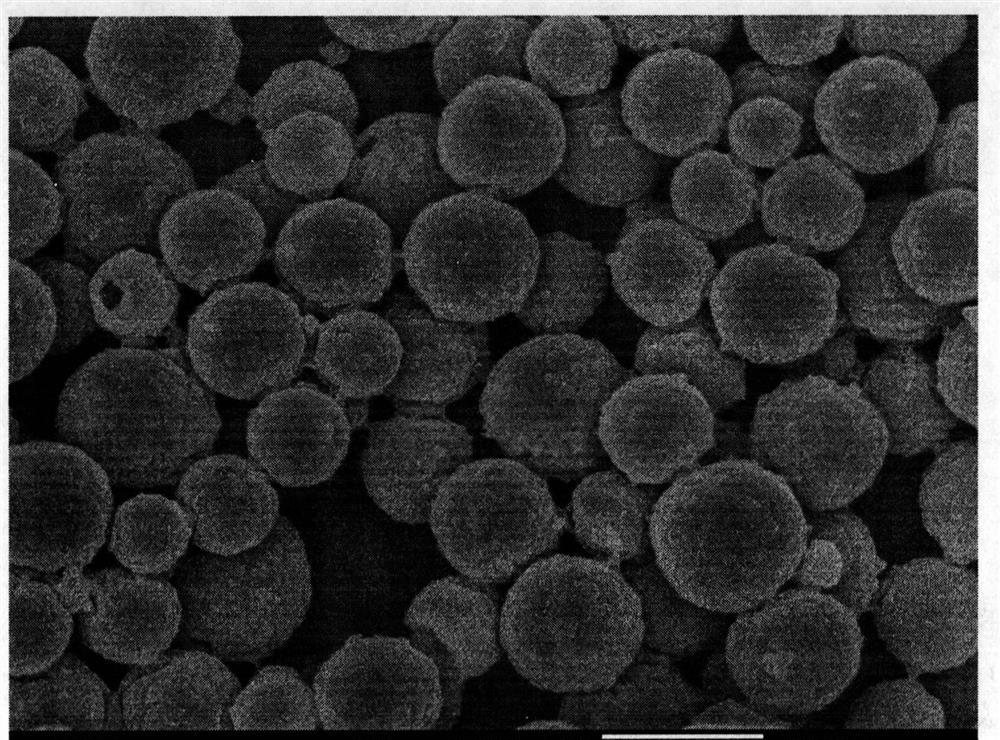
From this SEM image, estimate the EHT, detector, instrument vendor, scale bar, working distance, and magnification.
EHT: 5 kV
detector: SEI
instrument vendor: JEOL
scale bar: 1000 nm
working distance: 7.4 mm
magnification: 20 K X